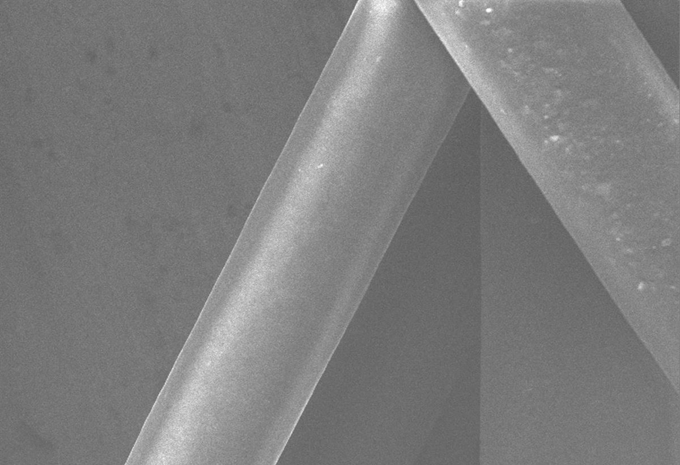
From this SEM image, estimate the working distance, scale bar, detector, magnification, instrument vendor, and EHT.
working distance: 0 mm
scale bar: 100000 nm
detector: SE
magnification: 0.5 K X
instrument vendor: JEOL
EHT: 10 kV